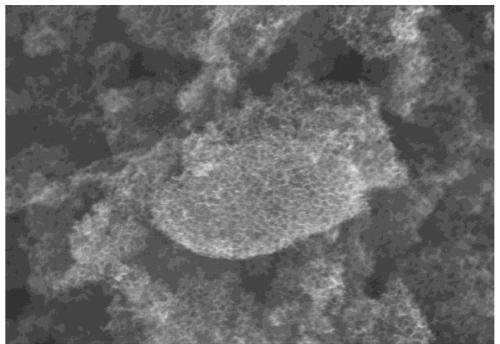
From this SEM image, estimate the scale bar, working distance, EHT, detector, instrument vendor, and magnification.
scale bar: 4000 nm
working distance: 8.4 mm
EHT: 15 kV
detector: SE(UL)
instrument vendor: Hitachi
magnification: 12 K X